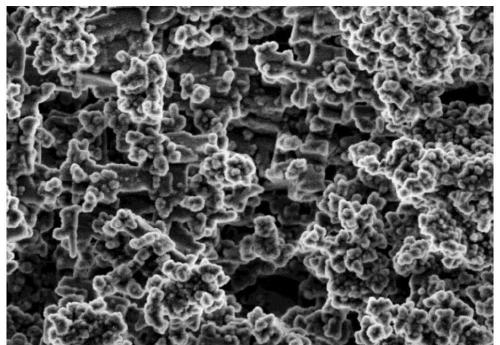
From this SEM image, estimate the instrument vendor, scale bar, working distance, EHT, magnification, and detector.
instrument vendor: Hitachi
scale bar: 10000 nm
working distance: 3.8 mm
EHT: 5 kV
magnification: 3 K X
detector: SE(M)